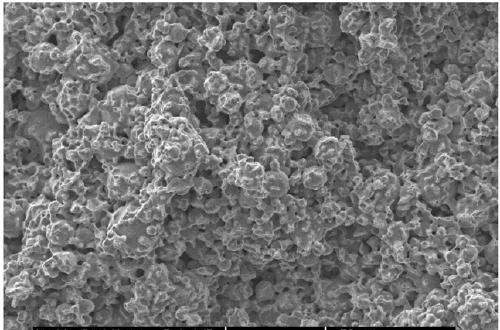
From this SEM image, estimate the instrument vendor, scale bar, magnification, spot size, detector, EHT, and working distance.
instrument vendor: FEI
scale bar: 50000 nm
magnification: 0.5 K X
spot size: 3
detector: SE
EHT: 15 kV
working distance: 5.9 mm